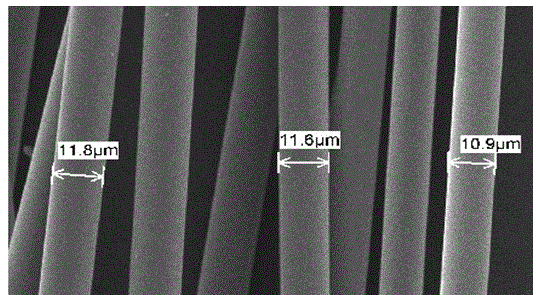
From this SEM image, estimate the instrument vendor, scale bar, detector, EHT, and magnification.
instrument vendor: JEOL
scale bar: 10000 nm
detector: SEI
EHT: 25 kV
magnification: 1 K X